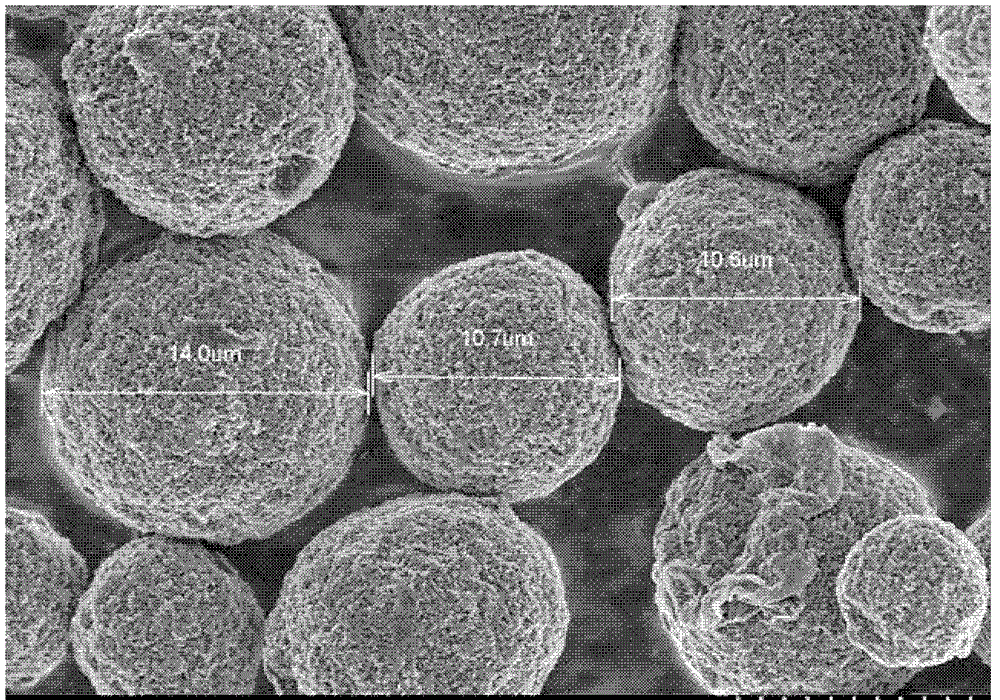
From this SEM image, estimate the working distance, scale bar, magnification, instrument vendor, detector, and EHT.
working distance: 8.1 mm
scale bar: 10000 nm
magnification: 3 K X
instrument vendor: Hitachi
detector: SE(M)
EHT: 3 kV